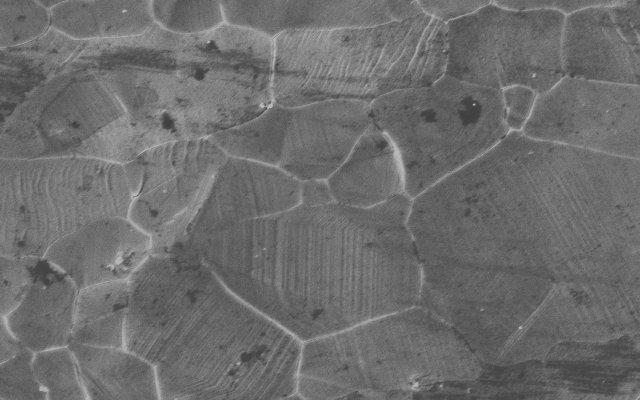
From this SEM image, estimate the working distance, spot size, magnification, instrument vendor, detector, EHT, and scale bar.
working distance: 17 mm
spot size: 4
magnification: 1.5 K X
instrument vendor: FEI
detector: SE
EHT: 15 kV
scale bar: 10000 nm